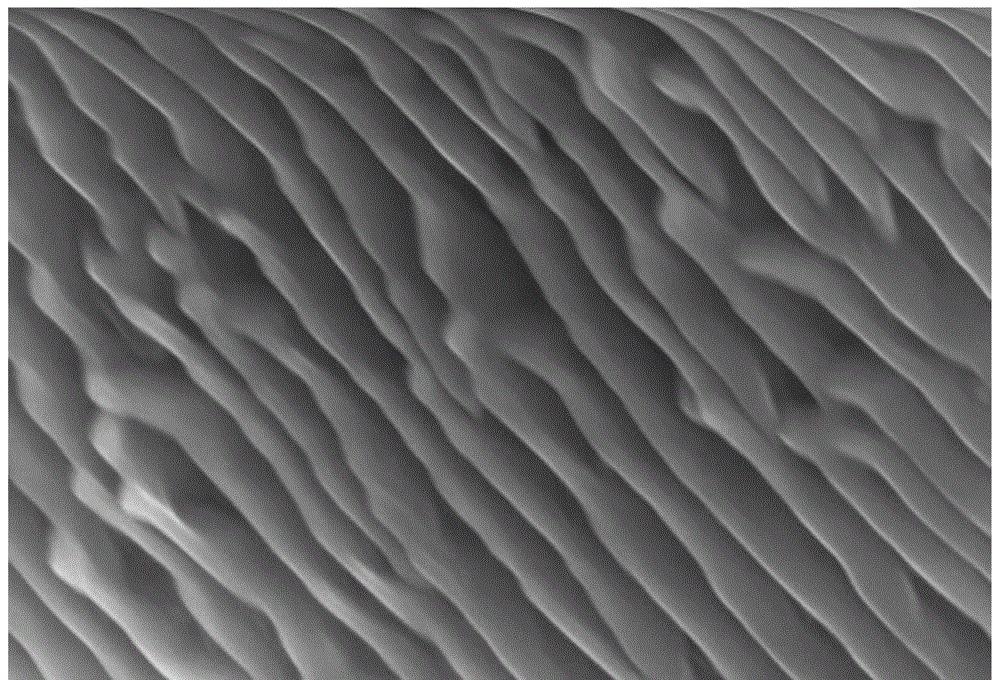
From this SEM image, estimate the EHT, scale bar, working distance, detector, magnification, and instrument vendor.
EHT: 15 kV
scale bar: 500 nm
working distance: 8.5 mm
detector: SE(U)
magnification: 100 K X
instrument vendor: Hitachi